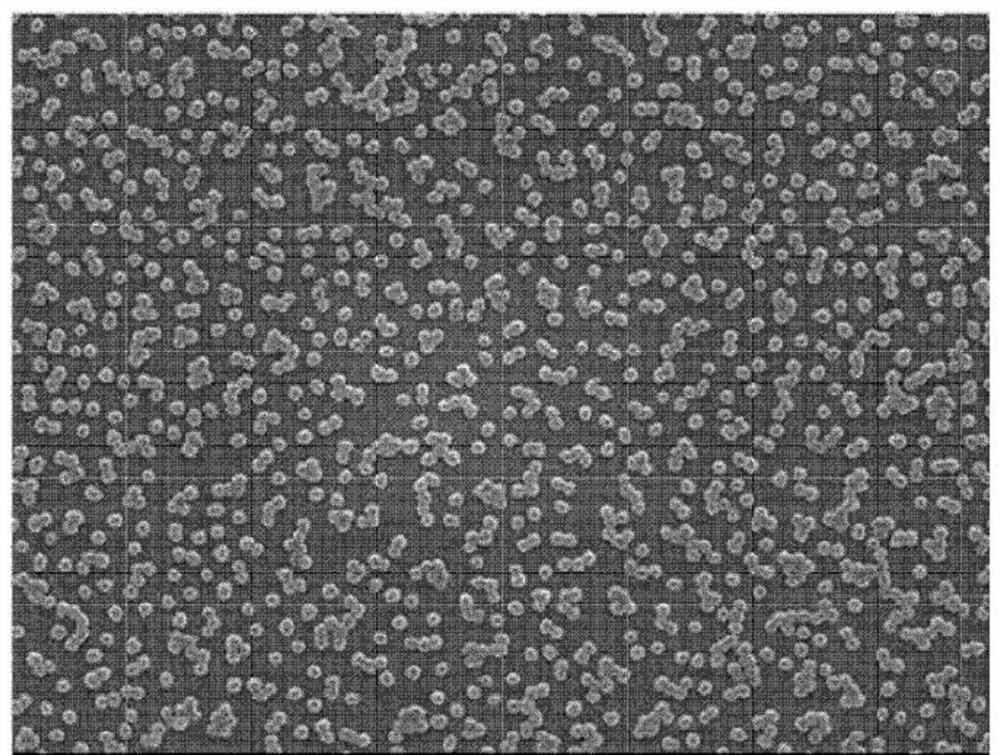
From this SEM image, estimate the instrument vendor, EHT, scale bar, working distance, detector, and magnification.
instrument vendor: JEOL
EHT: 5 kV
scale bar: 100 nm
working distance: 7.3 mm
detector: SEI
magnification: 30 K X